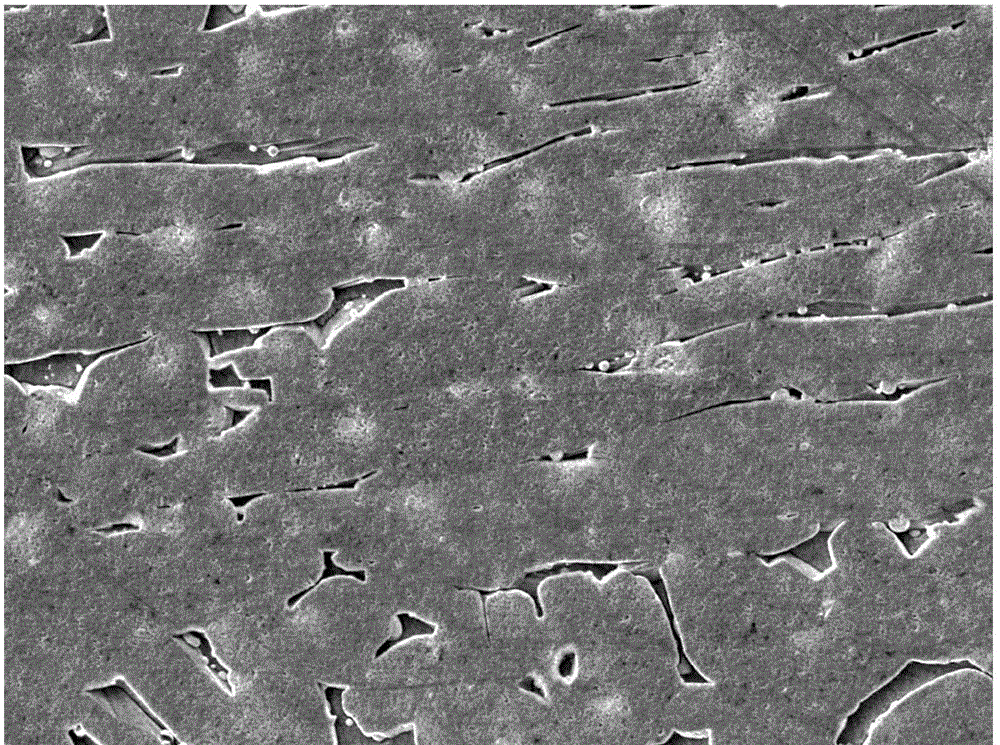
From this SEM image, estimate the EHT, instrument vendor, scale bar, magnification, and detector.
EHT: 20 kV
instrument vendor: JEOL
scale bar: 10000 nm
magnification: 0.5 K X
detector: LEI